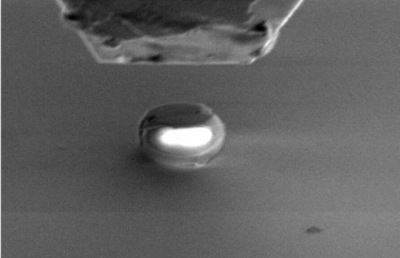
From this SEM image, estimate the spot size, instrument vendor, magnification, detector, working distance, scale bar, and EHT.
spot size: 3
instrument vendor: FEI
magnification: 5 K X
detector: SE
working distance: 10 mm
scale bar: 5000 nm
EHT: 3 kV